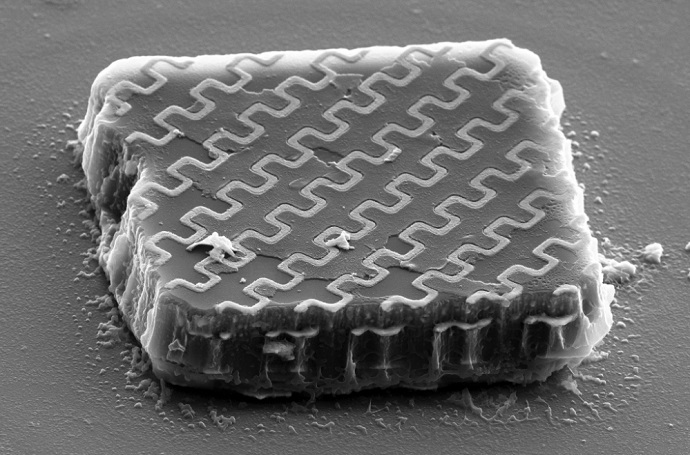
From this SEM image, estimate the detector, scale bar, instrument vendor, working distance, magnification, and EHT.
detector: SE2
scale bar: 1000 nm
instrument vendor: Zeiss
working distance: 10.9 mm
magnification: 10 K X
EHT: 10 kV